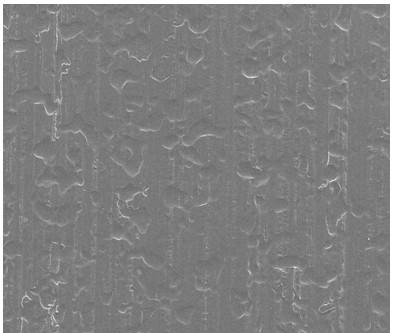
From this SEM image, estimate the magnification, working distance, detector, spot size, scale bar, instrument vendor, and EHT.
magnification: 0.25 K X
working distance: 9.7 mm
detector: ETD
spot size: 2.5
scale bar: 200000 nm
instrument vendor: FEI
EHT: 15 kV